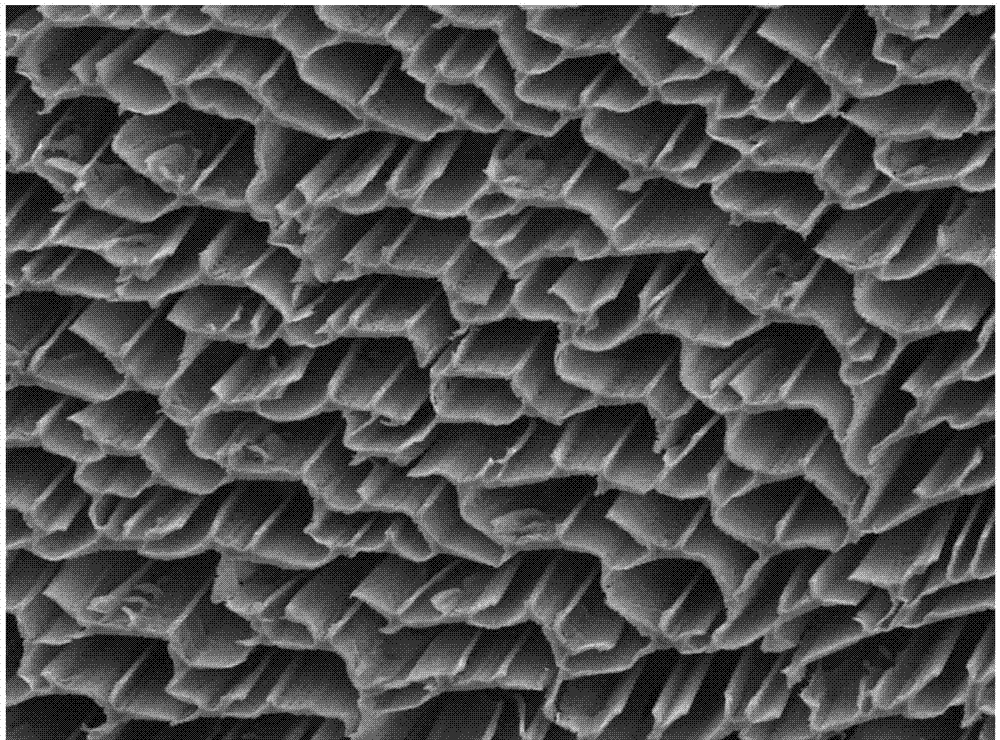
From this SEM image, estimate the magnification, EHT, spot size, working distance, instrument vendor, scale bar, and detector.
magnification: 0.15 K X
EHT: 20 kV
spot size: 30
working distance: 14.7 mm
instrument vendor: JEOL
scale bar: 100000 nm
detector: SED